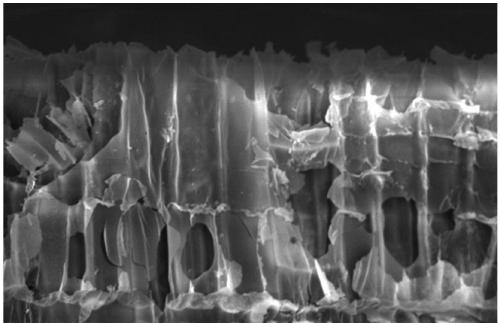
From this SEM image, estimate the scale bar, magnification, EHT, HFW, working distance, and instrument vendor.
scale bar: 50000 nm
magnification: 0.7 K X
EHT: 15 kV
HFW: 296 µm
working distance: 10.1 mm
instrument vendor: FEI